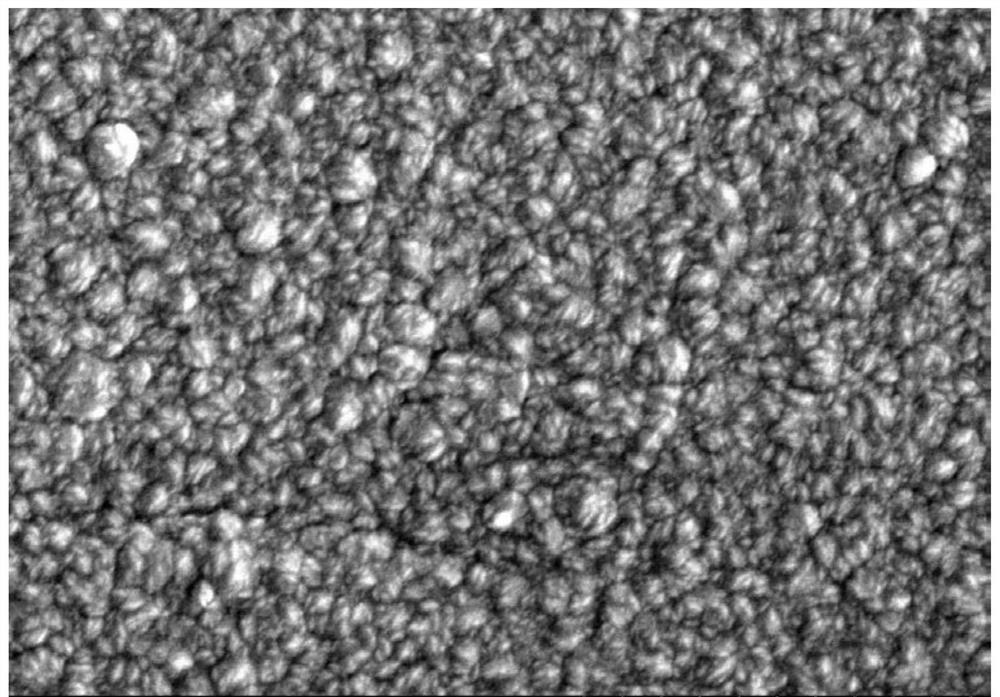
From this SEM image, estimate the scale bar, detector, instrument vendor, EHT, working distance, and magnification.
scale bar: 10000 nm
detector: SE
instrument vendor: Hitachi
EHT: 15 kV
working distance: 10.3 mm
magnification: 5 K X